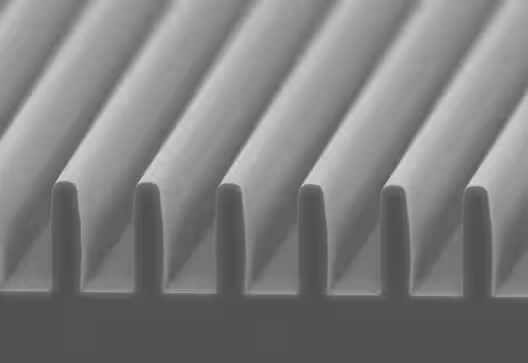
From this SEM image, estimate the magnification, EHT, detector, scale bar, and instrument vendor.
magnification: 10 K X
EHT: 5 kV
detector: SE(L)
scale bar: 5000 nm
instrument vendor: Hitachi